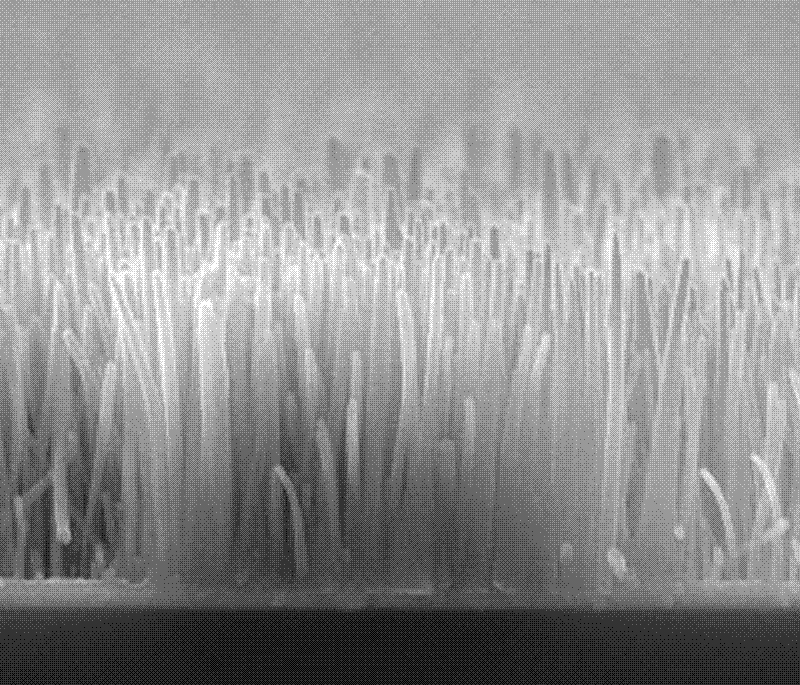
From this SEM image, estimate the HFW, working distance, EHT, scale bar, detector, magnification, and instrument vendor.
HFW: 7.46 µm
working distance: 7.7 mm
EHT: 15 kV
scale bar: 2000 nm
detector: ETD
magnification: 20 K X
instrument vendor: FEI